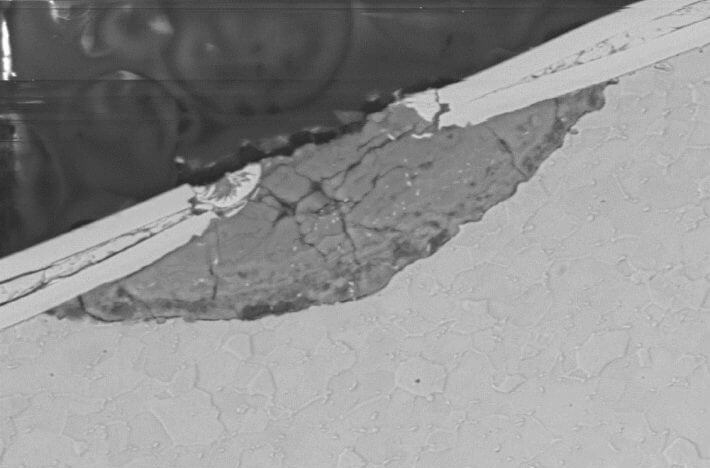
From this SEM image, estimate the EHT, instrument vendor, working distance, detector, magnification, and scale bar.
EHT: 20 kV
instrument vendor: Zeiss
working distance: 8.5 mm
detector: NTS BSD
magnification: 2 K X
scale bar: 20000 nm